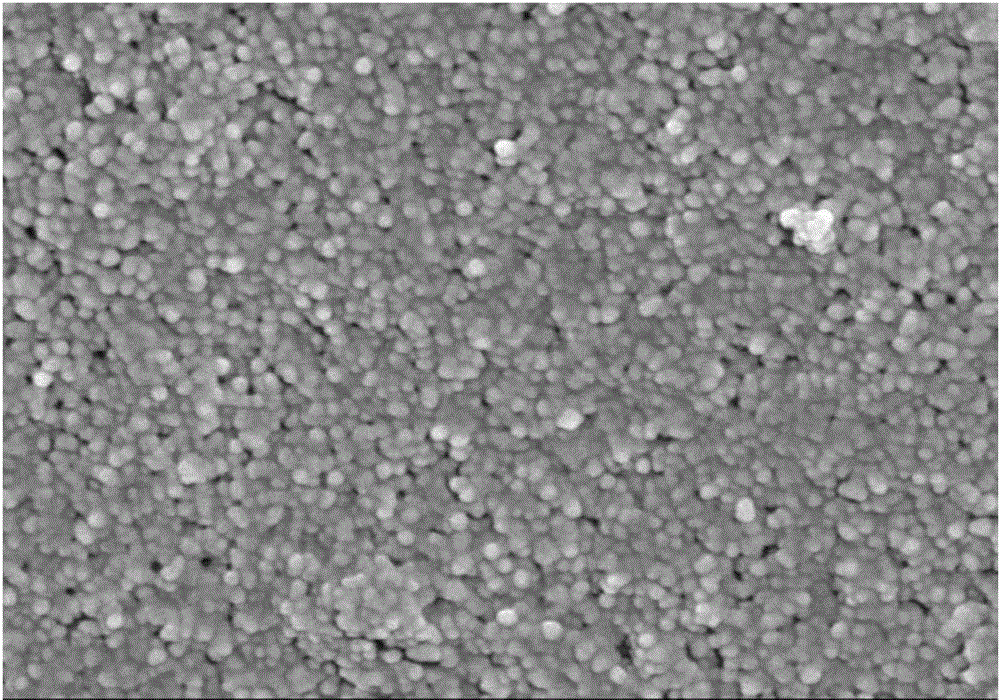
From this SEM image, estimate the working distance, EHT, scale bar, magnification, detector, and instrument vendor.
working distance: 7.9 mm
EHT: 5 kV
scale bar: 100 nm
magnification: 80 K X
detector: SEI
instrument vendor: JEOL